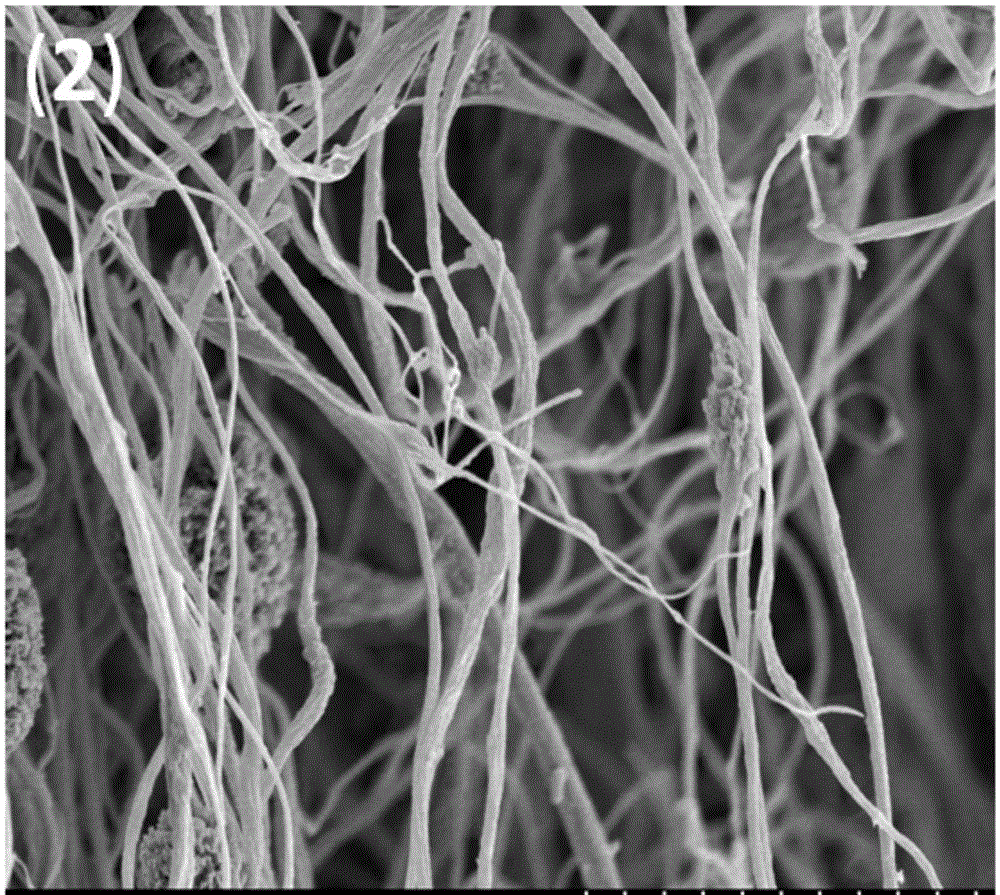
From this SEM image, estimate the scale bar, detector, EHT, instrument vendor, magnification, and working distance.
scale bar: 10000 nm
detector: SE(M)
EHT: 5 kV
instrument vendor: Hitachi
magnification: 5 K X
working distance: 7.8 mm